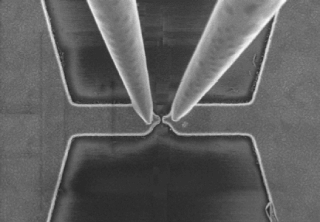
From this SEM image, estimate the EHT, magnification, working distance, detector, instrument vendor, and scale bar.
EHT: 2 kV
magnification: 30 K X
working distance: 5.4 mm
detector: SE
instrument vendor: Hitachi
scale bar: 1000 nm